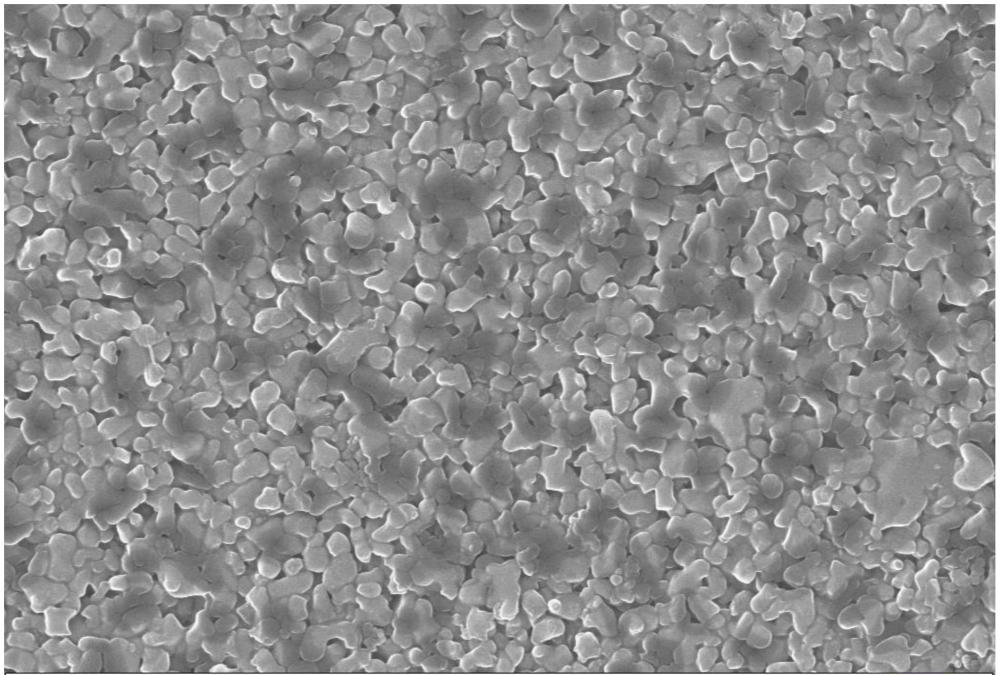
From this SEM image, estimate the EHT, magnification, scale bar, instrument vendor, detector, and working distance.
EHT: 20 kV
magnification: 10 K X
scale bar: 1000 nm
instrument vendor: Zeiss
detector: InLens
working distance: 3.8 mm